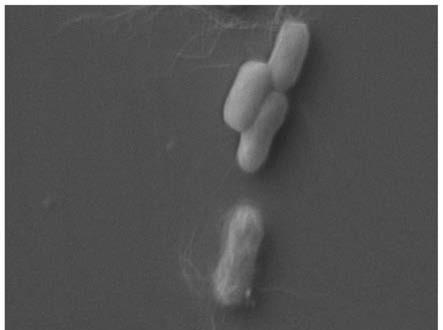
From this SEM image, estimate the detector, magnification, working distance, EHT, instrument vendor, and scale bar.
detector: SE1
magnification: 10 K X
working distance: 6.5 mm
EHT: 10 kV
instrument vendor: Zeiss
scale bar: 1000 nm